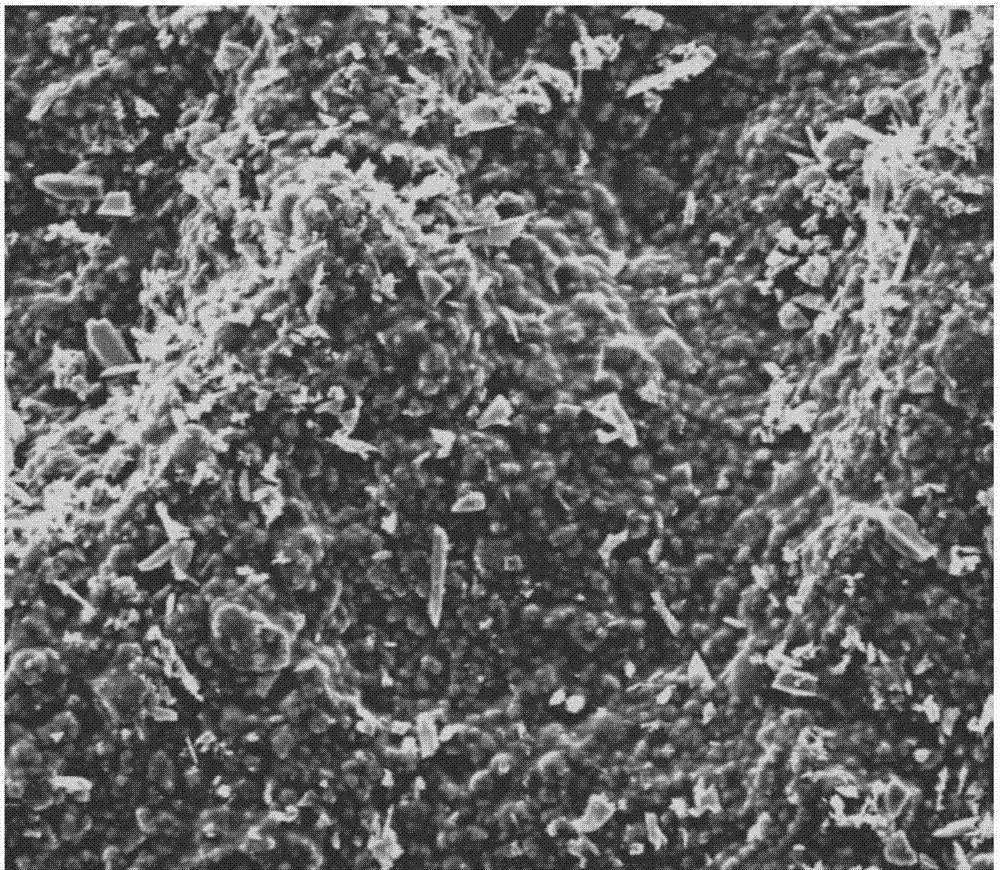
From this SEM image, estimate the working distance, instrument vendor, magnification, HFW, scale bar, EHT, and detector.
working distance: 11 mm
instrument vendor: FEI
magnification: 1.6 K X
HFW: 186 µm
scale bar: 50000 nm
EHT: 10 kV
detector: ETD